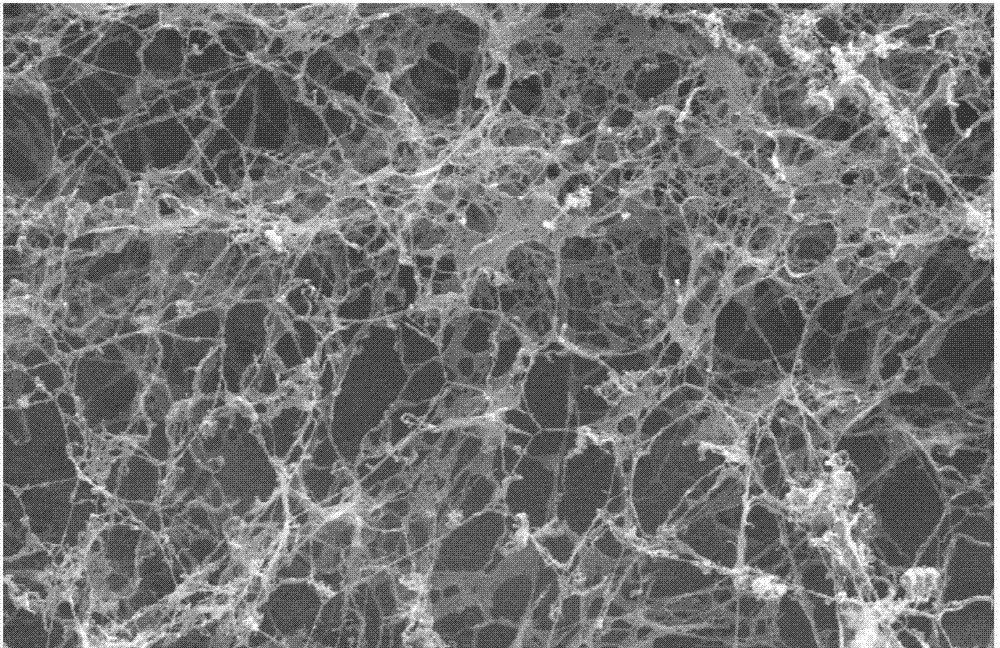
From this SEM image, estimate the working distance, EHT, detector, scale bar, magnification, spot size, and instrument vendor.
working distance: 6.8 mm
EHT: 25 kV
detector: SE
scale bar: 2000 nm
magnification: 20 K X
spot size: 4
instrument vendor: FEI